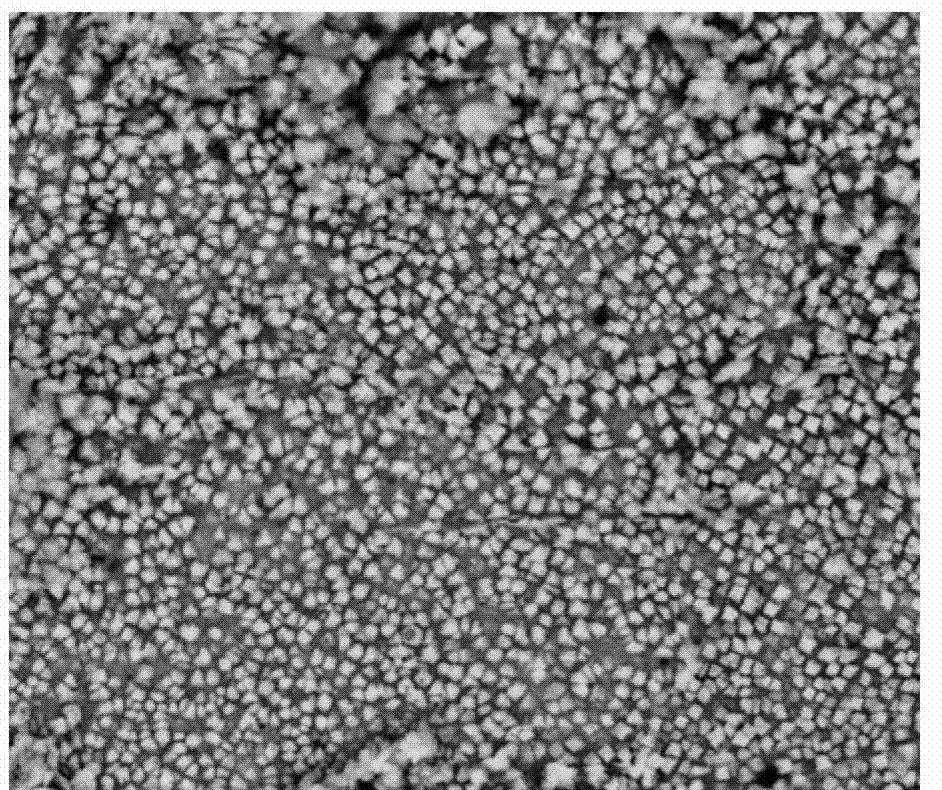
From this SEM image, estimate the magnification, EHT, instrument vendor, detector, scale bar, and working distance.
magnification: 10 K X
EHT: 25 kV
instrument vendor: FEI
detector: A+B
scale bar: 10000 nm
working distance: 10.6 mm